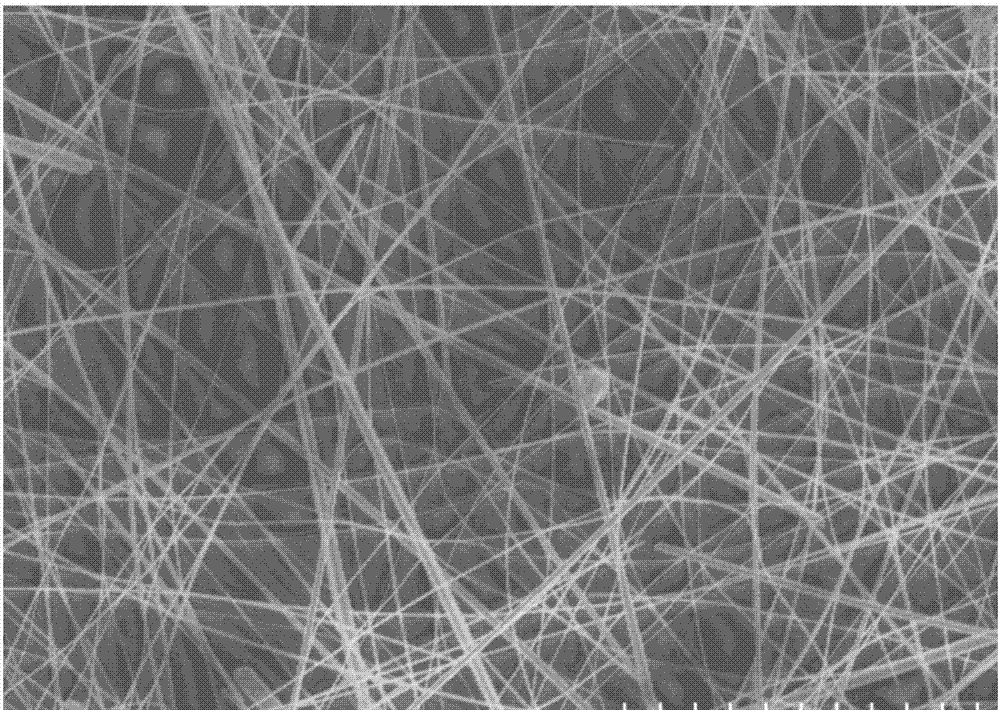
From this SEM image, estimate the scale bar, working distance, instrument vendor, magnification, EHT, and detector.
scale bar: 10000 nm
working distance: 10.4 mm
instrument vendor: Hitachi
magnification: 4.5 K X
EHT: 5 kV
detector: SE(M)